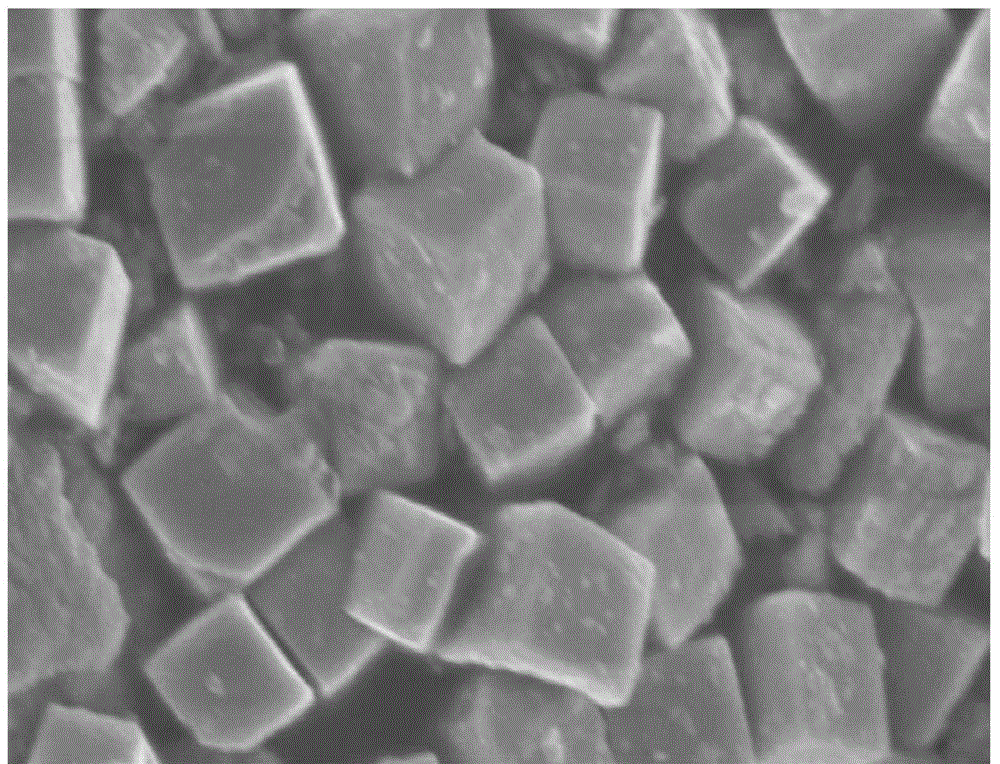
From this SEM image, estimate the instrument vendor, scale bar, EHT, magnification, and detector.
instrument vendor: Hitachi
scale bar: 1000 nm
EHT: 4 kV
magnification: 45 K X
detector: SE(M)